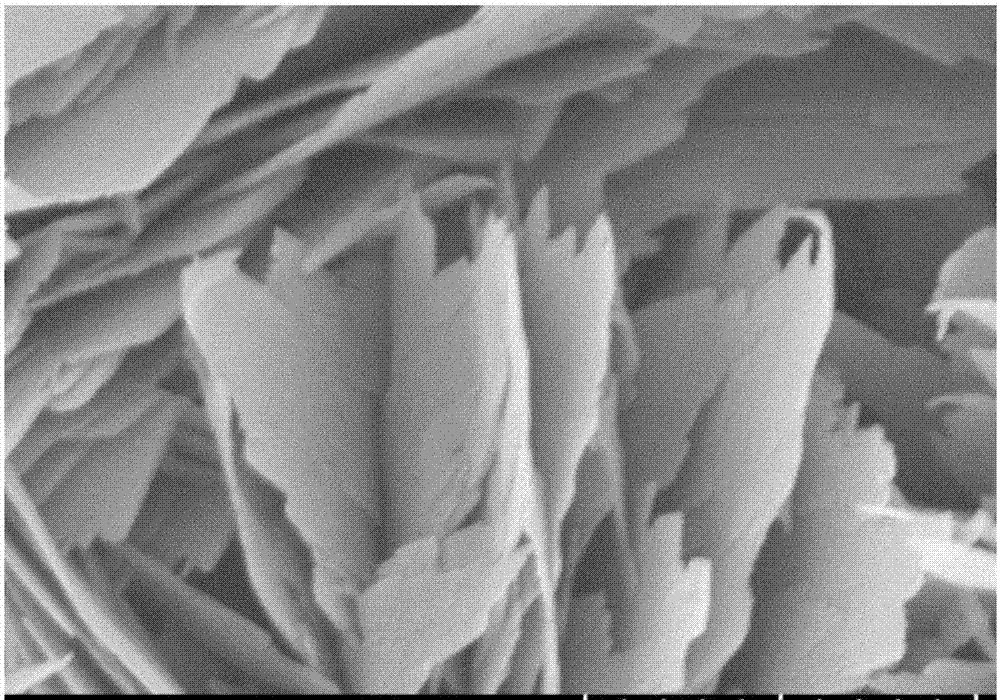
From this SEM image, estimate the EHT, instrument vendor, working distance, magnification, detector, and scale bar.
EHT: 5 kV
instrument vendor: Hitachi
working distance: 8.7 mm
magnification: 50 K X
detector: SE(UL)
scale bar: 1000 nm